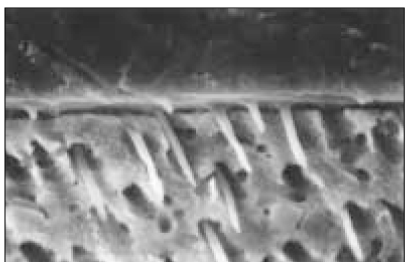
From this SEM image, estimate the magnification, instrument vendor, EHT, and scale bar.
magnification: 2 K X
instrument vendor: Hitachi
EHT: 20 kV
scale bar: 20000 nm